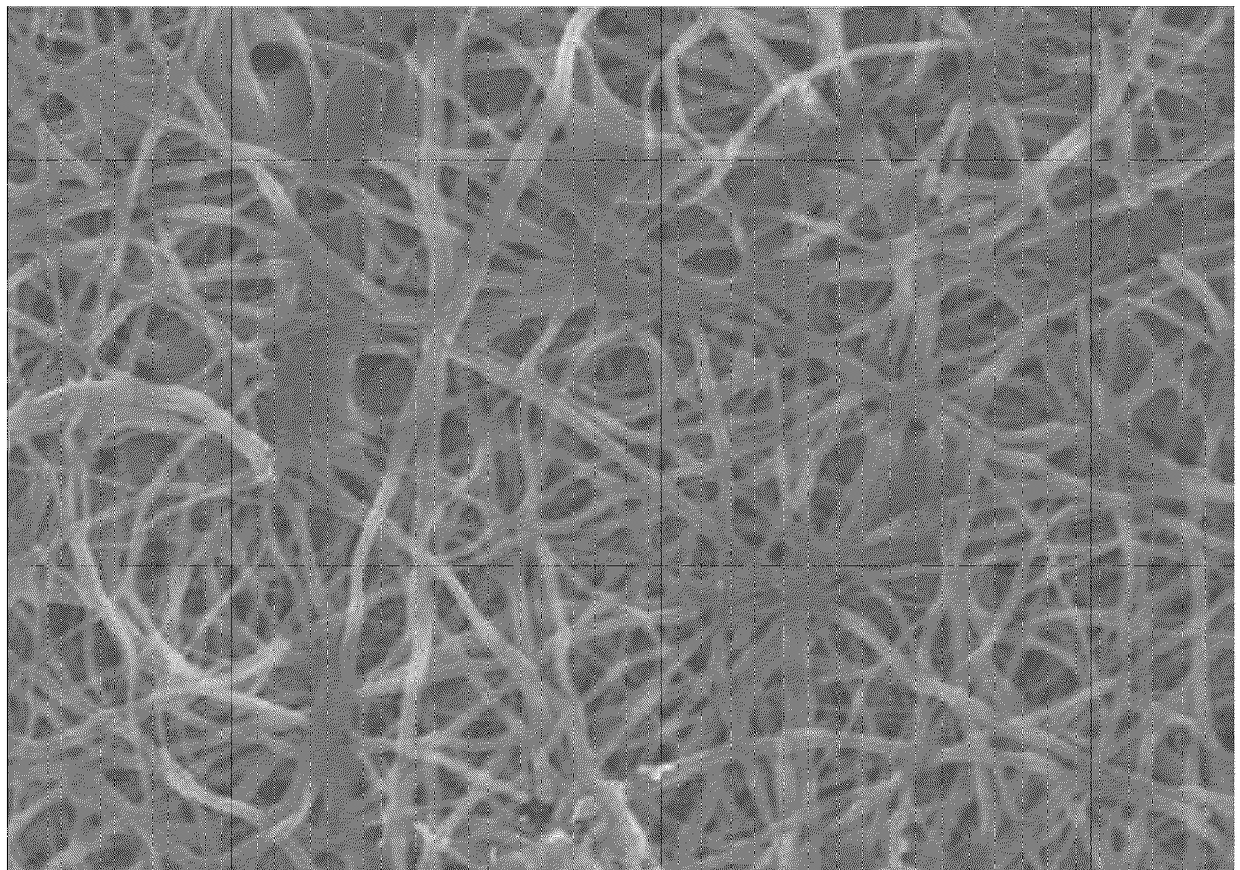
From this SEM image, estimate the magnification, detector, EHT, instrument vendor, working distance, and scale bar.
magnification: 2 K X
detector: SE(U)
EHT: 15 kV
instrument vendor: Hitachi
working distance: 8 mm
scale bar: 20000 nm